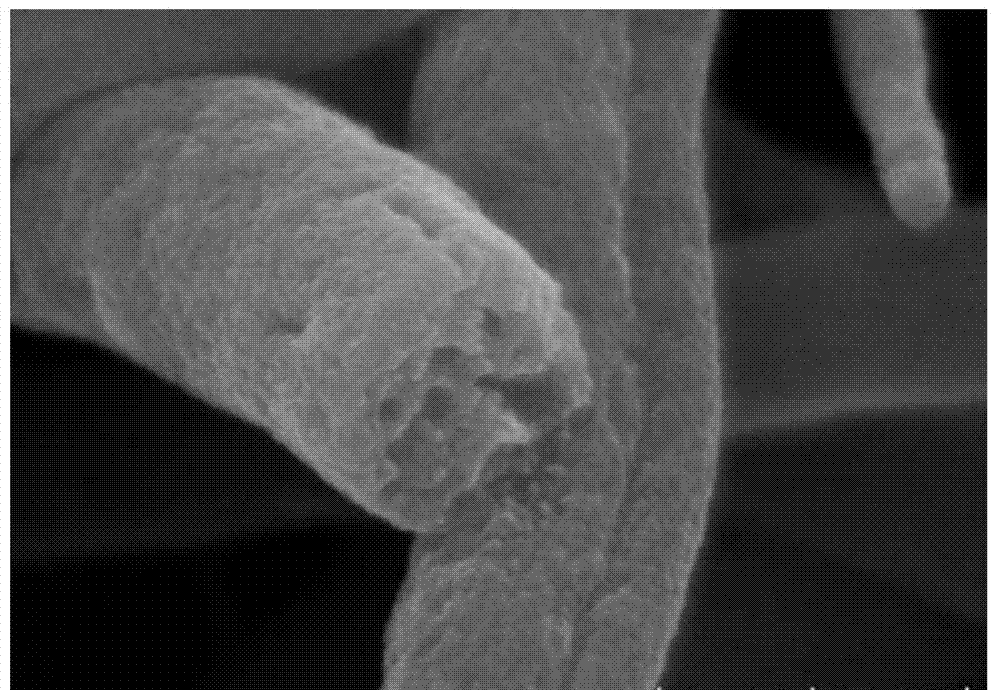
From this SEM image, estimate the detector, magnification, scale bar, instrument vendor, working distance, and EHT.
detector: SE(U)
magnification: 200 K X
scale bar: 200 nm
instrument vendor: Hitachi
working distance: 4 mm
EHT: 5 kV